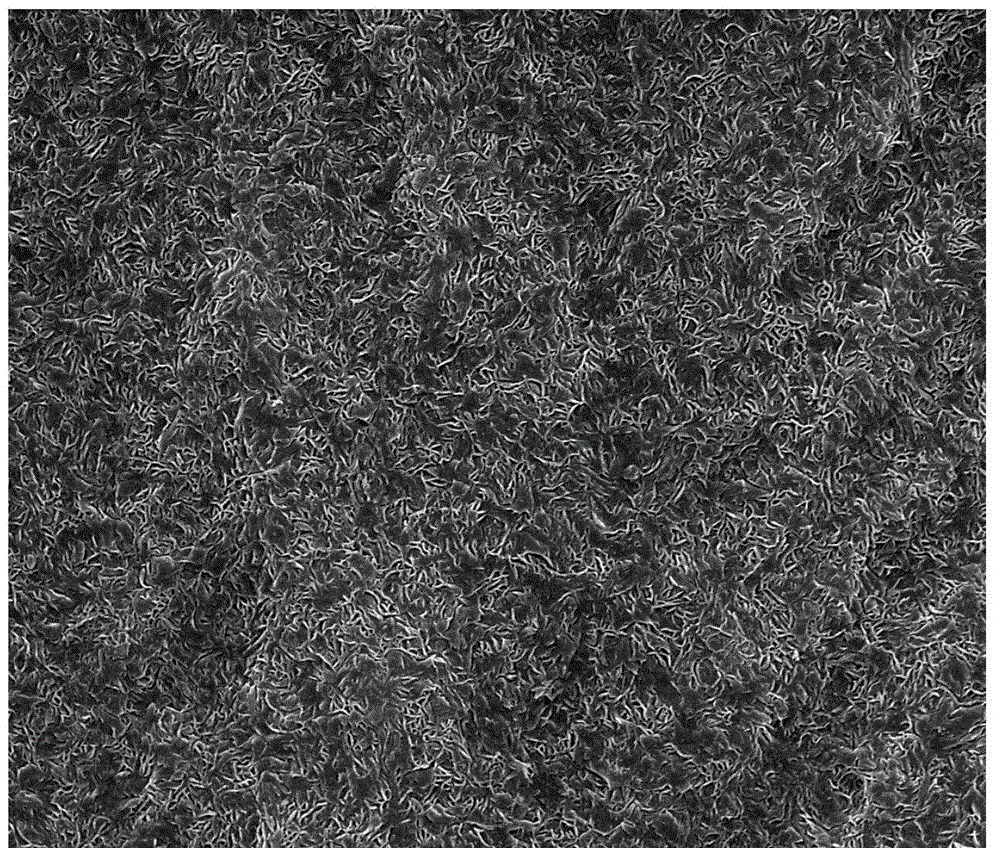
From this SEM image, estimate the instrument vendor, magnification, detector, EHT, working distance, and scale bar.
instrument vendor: FEI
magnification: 10 K X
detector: SE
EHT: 20 kV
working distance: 10.8 mm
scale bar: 10000 nm